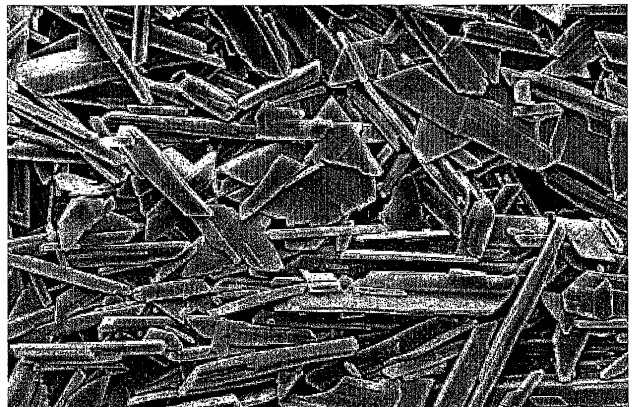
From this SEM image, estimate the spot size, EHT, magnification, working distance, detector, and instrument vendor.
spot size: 2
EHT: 3 kV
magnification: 10 K X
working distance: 10 mm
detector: SE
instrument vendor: FEI